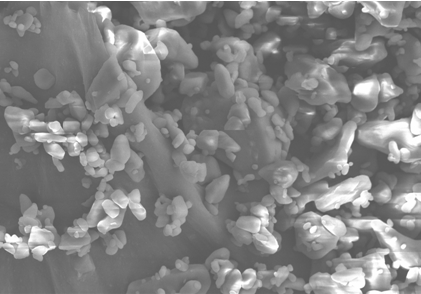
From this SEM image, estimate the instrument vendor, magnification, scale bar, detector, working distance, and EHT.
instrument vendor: Hitachi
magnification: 1 K X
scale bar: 50000 nm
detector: SE(M)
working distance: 11.8 mm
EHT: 15 kV